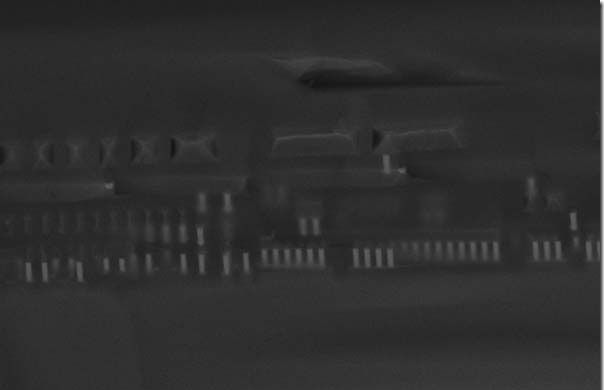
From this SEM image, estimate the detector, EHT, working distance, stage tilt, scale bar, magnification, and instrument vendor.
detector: InLens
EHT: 15 kV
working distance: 4 mm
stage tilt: -15°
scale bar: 2000 nm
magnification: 11.47 K X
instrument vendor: Zeiss